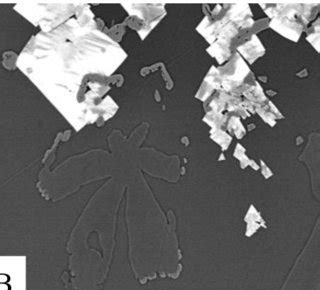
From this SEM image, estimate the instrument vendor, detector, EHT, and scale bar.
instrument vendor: JEOL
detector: COMP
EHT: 10 kV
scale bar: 10000 nm